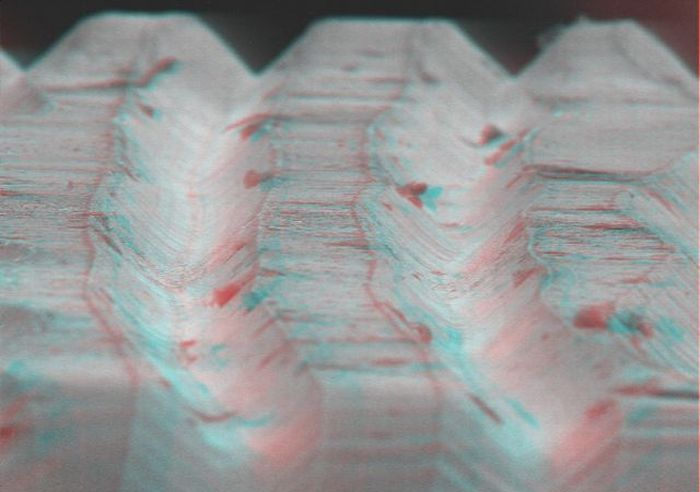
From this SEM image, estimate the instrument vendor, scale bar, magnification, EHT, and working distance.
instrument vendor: other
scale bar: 100000 nm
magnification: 0.39 K X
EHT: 15 kV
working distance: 30 mm